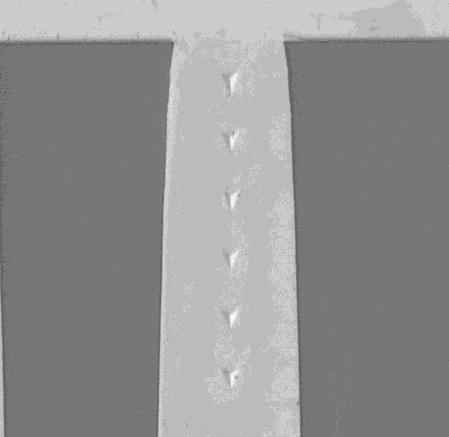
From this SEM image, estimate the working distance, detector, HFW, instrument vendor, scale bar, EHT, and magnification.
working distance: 45.68 mm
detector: SE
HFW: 151 µm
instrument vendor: Tescan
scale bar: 20000 nm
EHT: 10 kV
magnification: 3.66 K X